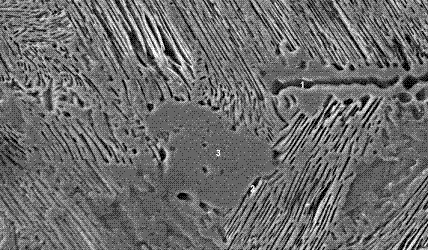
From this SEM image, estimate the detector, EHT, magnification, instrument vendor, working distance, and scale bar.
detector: SE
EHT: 20 kV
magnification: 2 K X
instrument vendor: FEI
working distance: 11.3 mm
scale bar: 10000 nm